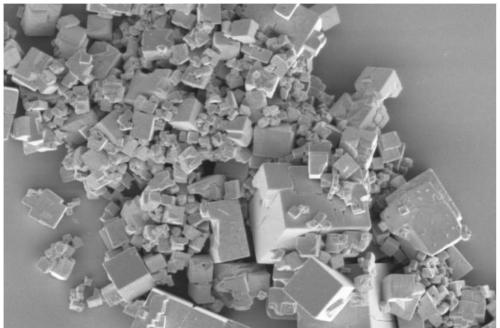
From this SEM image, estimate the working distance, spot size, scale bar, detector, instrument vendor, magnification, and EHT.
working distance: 3 mm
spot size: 4.5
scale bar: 20000 nm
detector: ETD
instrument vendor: FEI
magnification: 10 K X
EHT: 3 kV